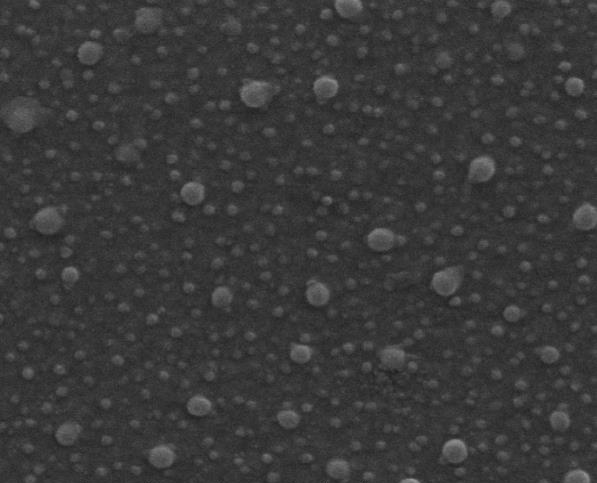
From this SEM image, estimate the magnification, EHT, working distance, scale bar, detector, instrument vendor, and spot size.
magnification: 160 K X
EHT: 20 kV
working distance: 10.5 mm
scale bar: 500 nm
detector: ETD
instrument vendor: FEI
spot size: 3